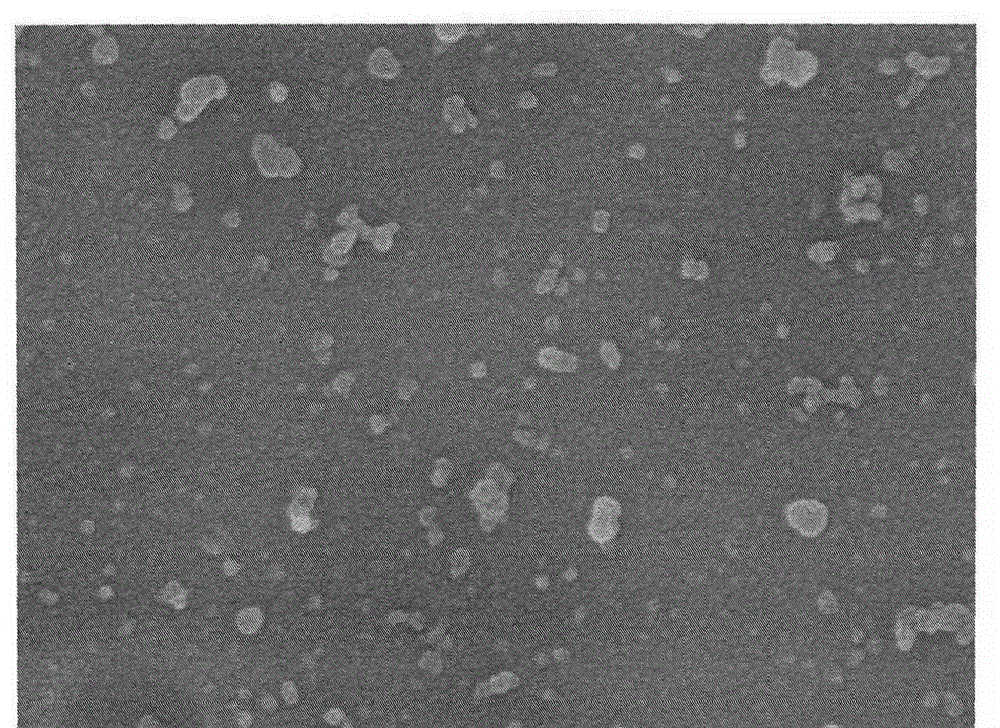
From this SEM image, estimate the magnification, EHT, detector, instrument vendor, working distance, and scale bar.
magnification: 40 K X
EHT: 7 kV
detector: SE(M)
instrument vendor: Hitachi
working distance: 9.5 mm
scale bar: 1000 nm